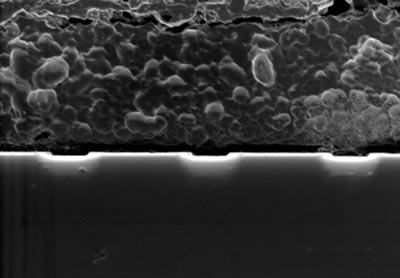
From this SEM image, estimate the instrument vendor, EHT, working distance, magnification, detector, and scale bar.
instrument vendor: Hitachi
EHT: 1 kV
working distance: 6.6 mm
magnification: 10 K X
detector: SE(U)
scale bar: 5000 nm